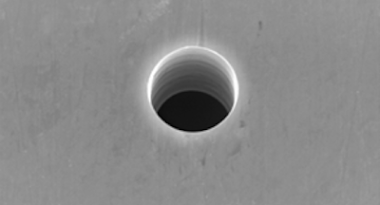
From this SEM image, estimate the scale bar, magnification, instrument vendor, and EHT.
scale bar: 5000 nm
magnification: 5 K X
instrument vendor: JEOL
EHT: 25 kV